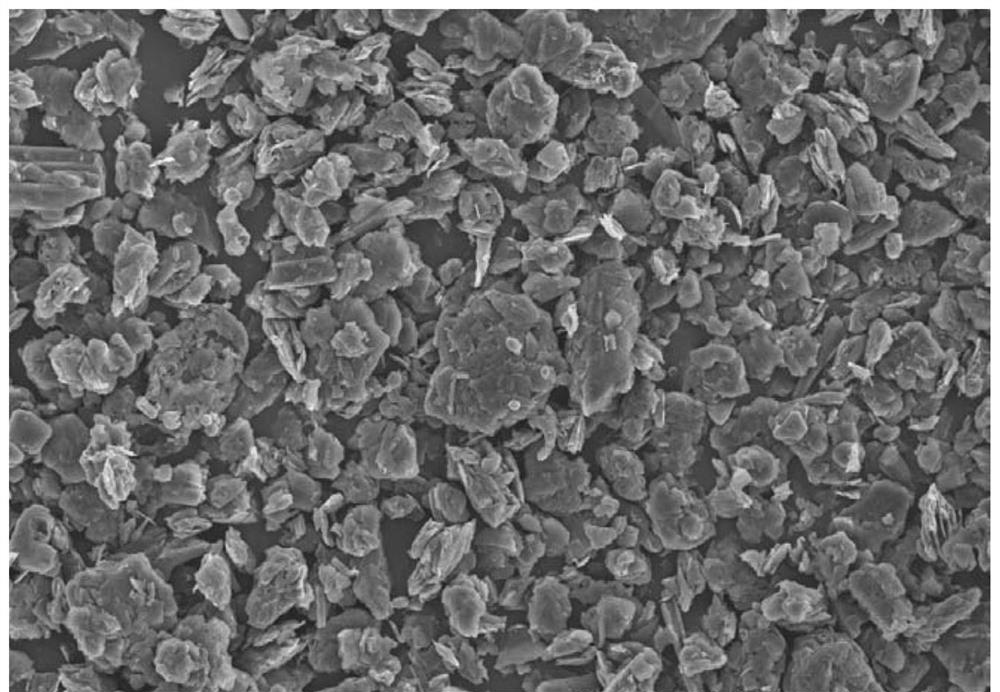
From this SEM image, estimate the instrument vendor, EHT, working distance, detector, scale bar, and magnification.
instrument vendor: Hitachi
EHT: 15 kV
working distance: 9.2 mm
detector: SE(UL)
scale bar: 100000 nm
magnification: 0.5 K X